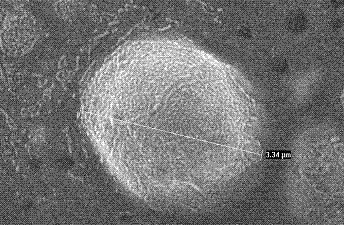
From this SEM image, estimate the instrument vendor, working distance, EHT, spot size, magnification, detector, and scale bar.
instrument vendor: FEI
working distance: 6.1 mm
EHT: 20 kV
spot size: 1.9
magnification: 20 K X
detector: SE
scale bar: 1000 nm